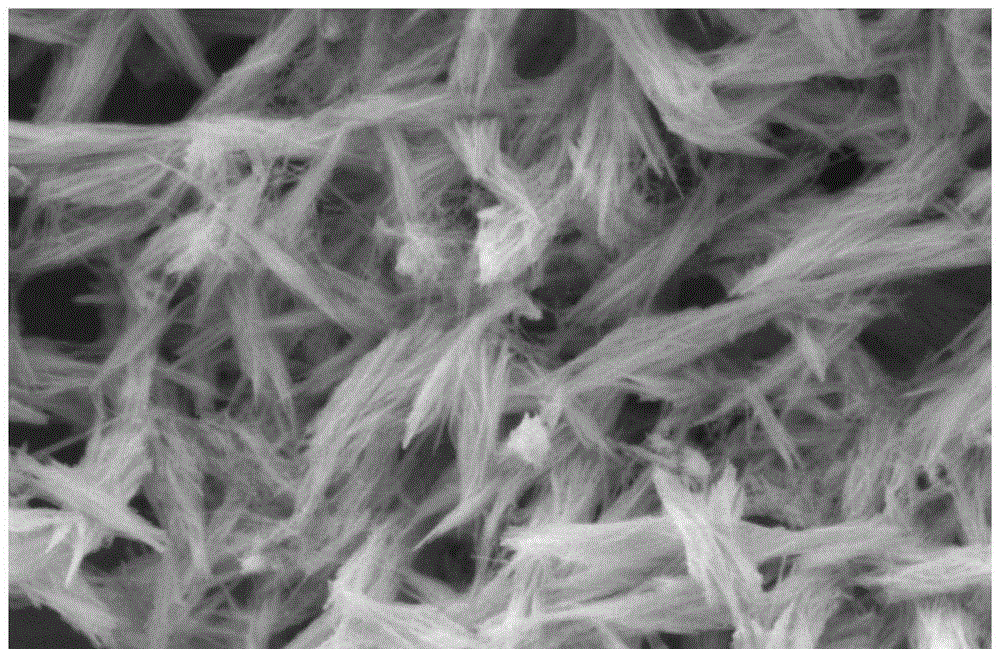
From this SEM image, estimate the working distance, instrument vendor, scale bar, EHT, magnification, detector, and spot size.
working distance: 4.6 mm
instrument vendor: FEI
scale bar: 2000 nm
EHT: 5 kV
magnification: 29.501 K X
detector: SE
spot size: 4